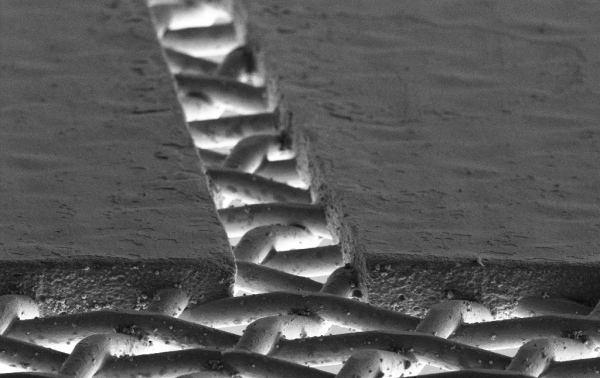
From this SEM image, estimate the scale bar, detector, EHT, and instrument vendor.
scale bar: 20000 nm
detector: SE2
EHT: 5 kV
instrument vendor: Zeiss